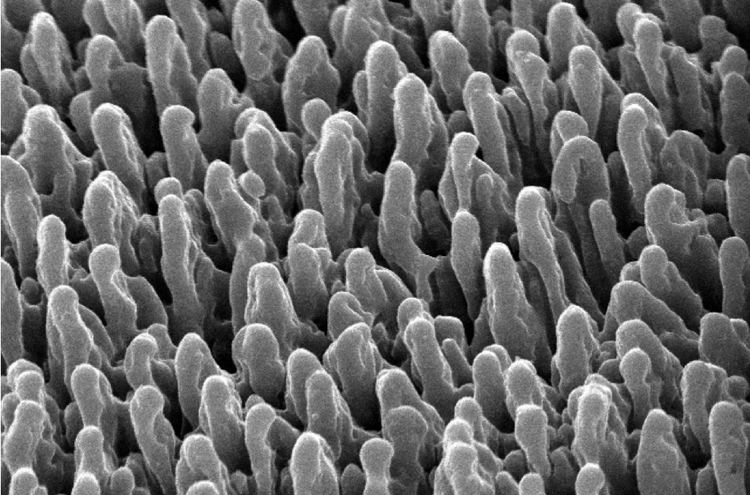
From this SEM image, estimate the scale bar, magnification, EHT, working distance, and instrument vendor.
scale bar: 1000 nm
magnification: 4 K X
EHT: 15 kV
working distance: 19 mm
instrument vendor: JEOL